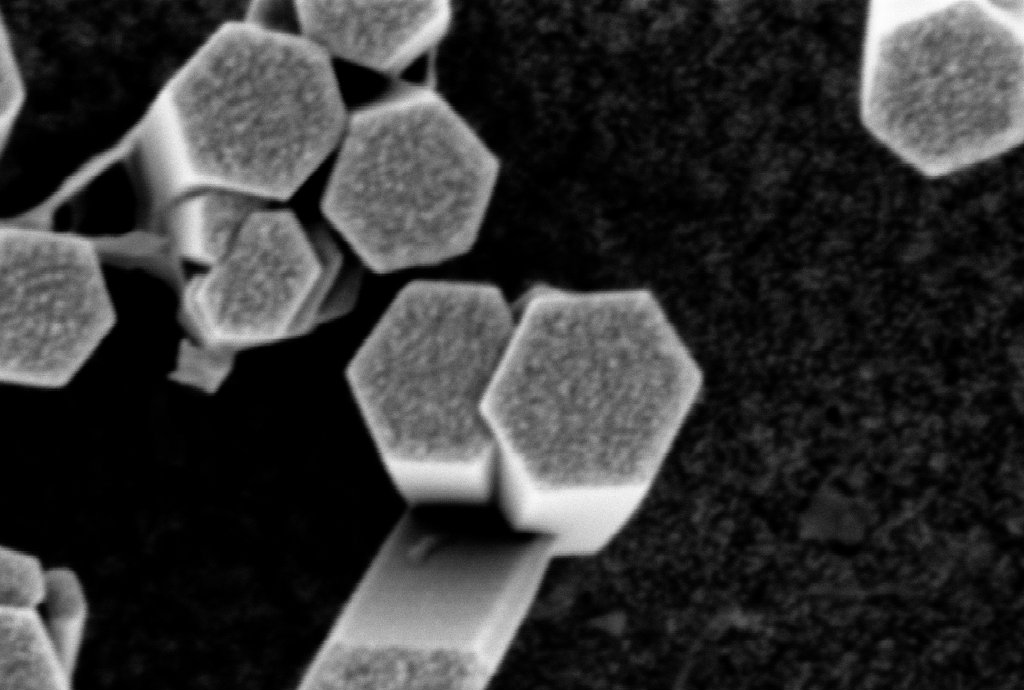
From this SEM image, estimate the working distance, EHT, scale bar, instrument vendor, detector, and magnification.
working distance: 5.3 mm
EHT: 1 kV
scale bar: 100 nm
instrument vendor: Zeiss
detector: InLens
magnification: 286.28 K X